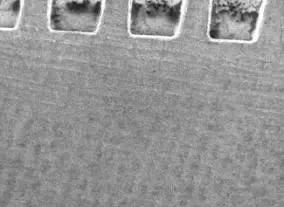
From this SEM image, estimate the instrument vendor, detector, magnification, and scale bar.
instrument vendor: JEOL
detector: SEI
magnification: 0.0117 K X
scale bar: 2000000 nm